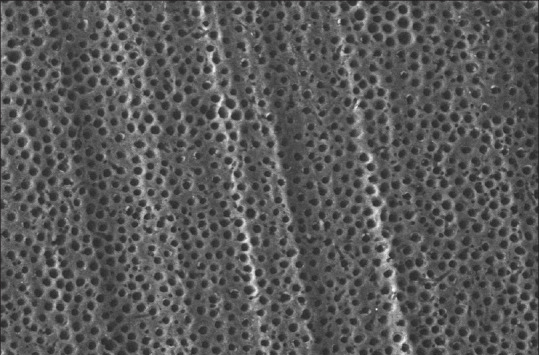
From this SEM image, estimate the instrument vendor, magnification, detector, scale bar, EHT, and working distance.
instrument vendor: Zeiss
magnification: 1.5 K X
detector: VPSE G3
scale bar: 10000 nm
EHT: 15 kV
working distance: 5.5 mm